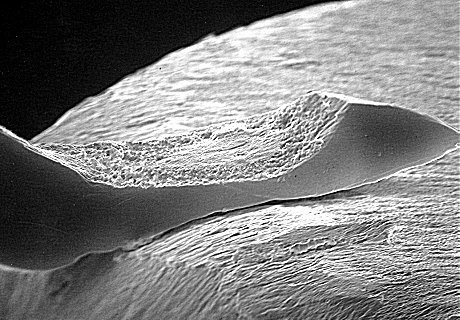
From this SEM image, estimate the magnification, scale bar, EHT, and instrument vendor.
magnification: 1.34 K X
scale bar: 10000 nm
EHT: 30 kV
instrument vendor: JEOL